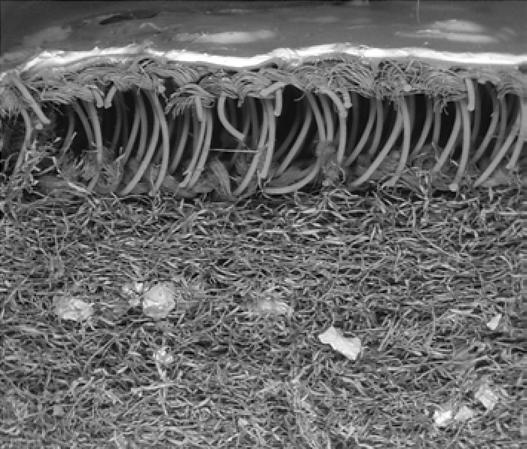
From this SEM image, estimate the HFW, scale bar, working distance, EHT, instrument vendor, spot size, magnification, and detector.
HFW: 7790 µm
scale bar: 3000000 nm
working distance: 24.6 mm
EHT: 20 kV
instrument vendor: FEI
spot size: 5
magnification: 0.038 K X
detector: BSED